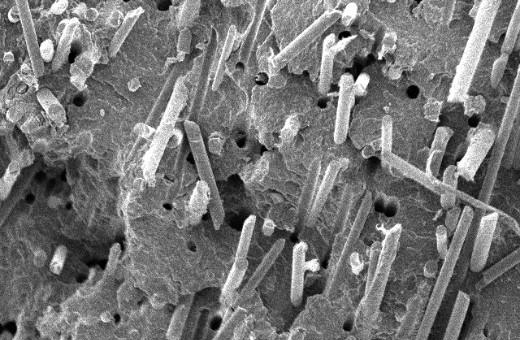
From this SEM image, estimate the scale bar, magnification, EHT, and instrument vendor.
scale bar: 100000 nm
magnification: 0.25 K X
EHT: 10 kV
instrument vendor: JEOL